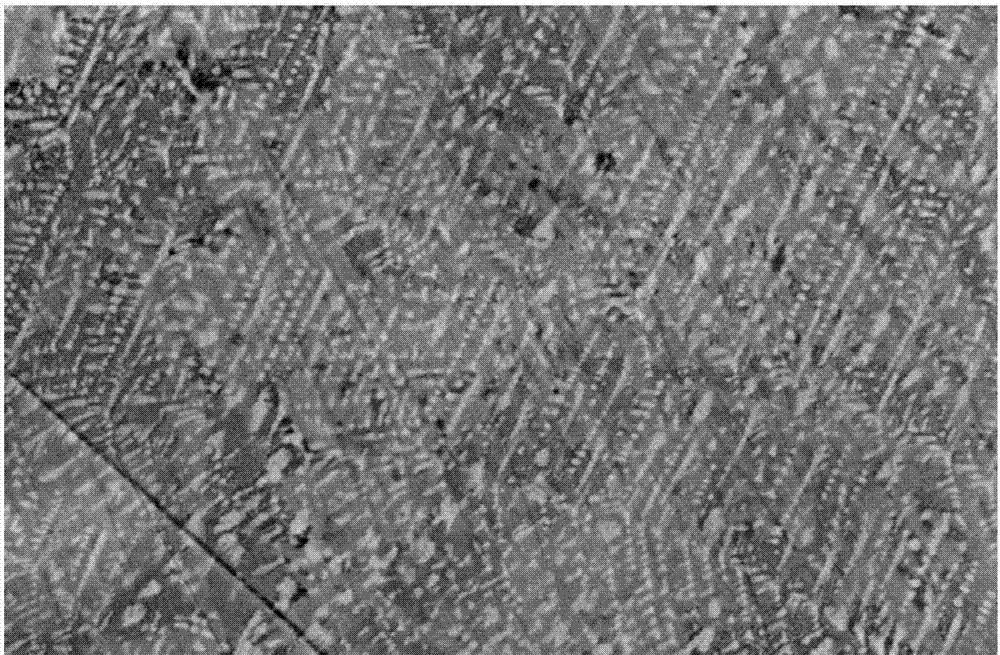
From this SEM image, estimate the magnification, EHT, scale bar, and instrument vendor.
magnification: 1 K X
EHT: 20 kV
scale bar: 10000 nm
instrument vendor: JEOL